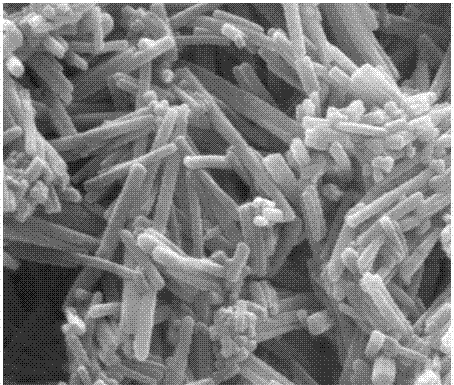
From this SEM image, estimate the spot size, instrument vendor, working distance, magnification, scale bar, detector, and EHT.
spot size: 2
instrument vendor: FEI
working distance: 10.1 mm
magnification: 60 K X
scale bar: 2000 nm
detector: ETD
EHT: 25 kV